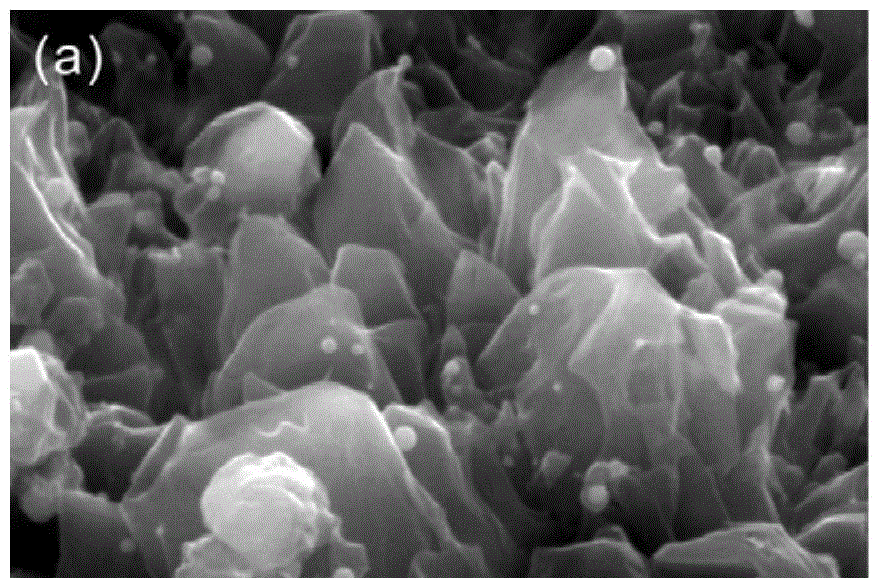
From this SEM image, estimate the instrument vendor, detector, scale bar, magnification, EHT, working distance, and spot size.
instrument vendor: FEI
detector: SE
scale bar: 500 nm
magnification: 40 K X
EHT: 15 kV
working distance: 20.6 mm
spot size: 3